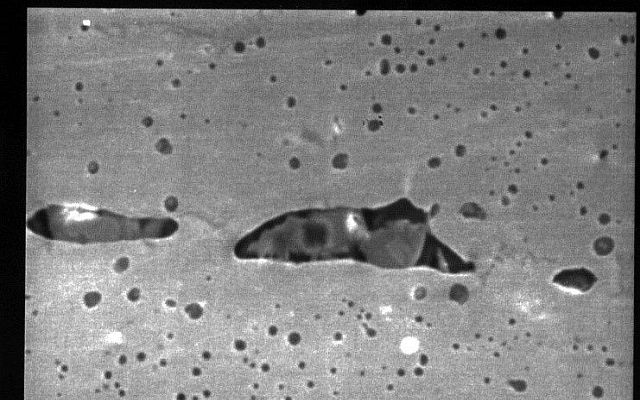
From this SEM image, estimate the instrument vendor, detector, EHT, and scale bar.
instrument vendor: JEOL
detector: SEI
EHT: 20 kV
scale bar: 10000 nm